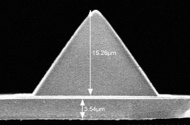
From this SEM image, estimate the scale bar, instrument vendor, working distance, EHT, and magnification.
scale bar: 10000 nm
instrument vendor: Hitachi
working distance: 6.9 mm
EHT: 15 kV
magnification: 3.54 K X